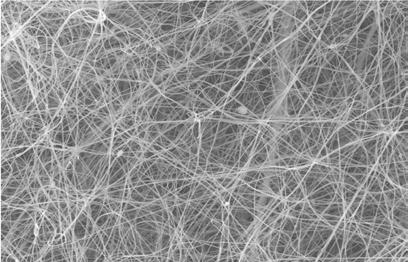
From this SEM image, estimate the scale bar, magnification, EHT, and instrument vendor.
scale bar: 10000 nm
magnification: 1 K X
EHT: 15 kV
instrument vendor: JEOL